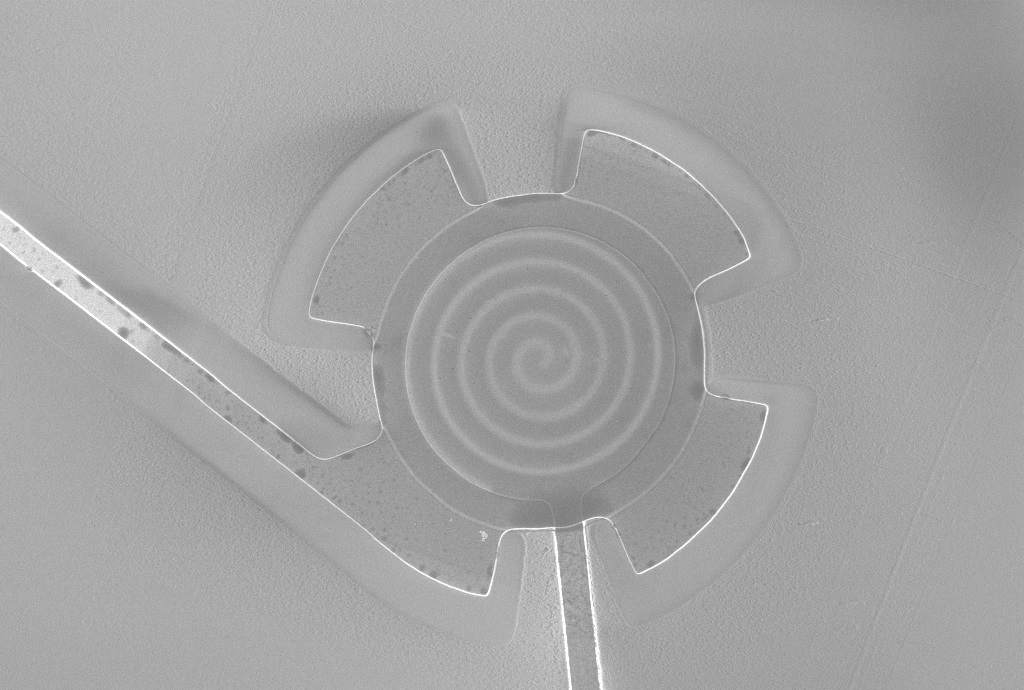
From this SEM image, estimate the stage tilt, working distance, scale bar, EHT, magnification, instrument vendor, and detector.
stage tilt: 0°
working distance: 4.6 mm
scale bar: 10000 nm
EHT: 2 kV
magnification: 1.29 K X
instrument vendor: Zeiss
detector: InLens